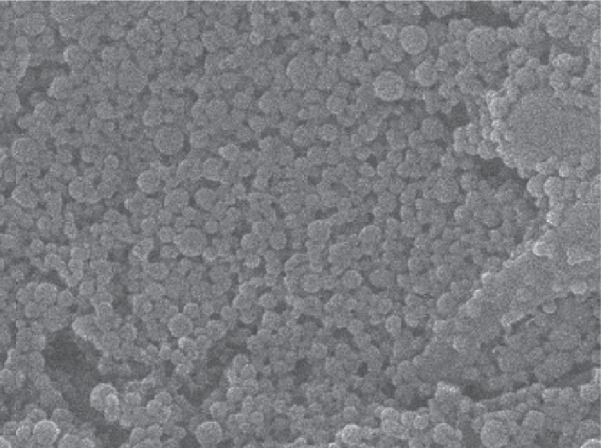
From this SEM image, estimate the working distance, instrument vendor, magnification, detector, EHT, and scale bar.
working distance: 8 mm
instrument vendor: JEOL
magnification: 30 K X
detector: LEI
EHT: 15 kV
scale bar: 100 nm